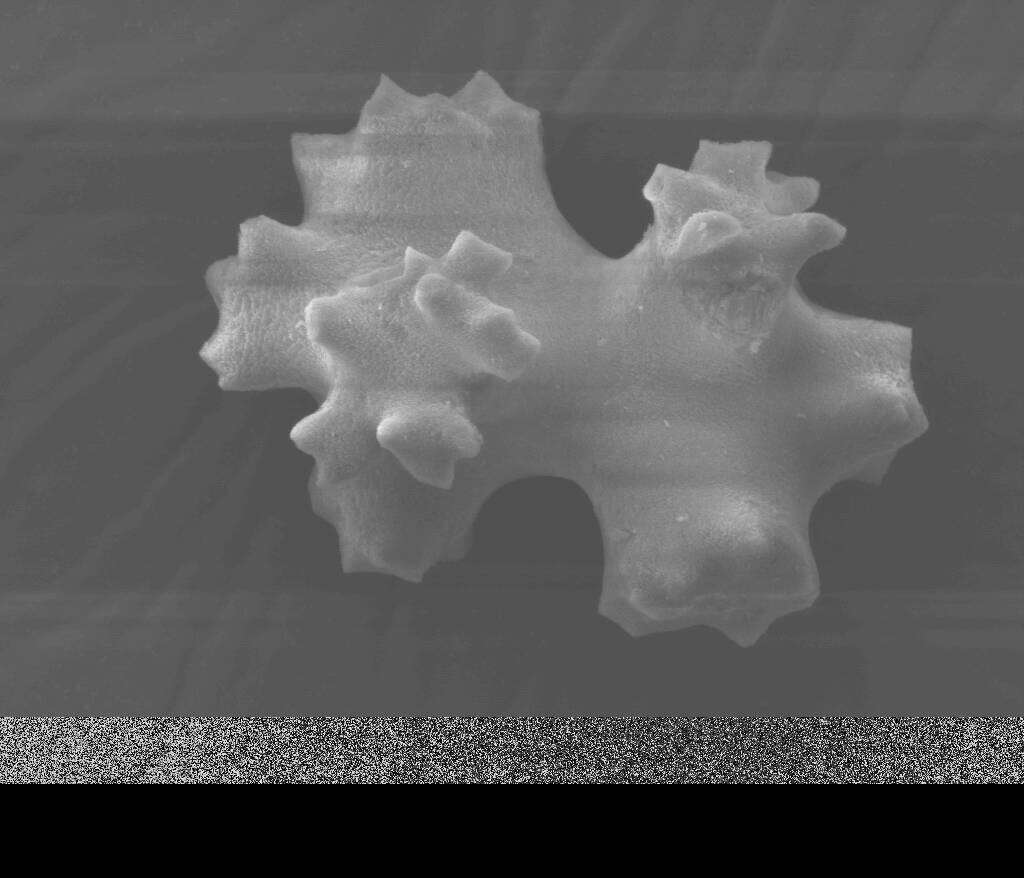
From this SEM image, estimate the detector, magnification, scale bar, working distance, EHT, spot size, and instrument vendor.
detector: SE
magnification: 3 K X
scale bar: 20000 nm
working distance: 20.1 mm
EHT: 12.5 kV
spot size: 3.5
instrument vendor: FEI